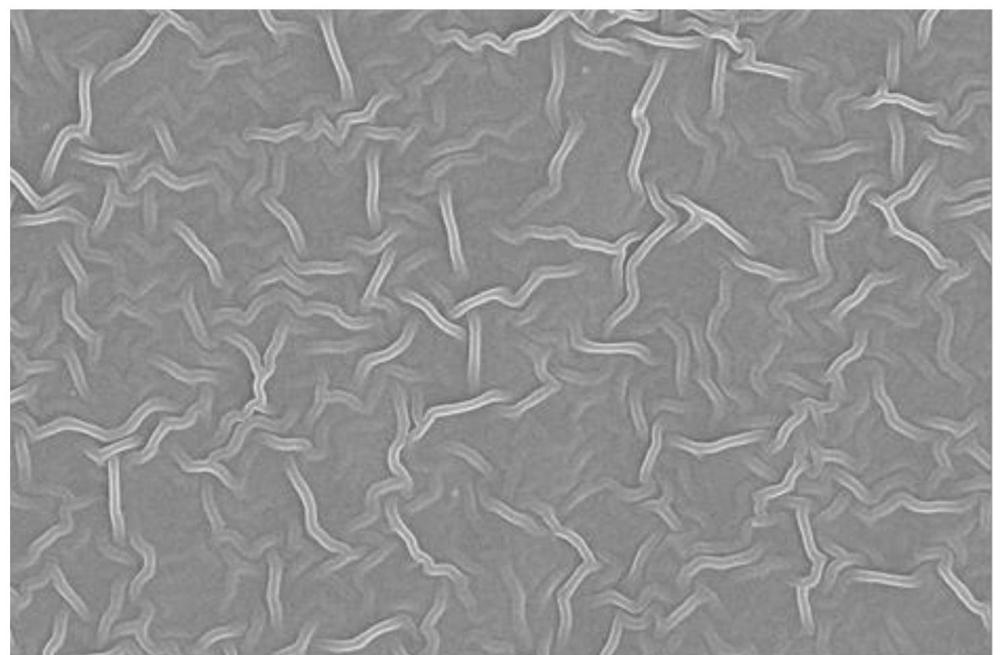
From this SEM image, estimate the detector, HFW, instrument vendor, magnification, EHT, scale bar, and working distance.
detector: T2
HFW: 12.7 µm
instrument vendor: Thermo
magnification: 10 K X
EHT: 20 kV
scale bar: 4000 nm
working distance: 10 mm